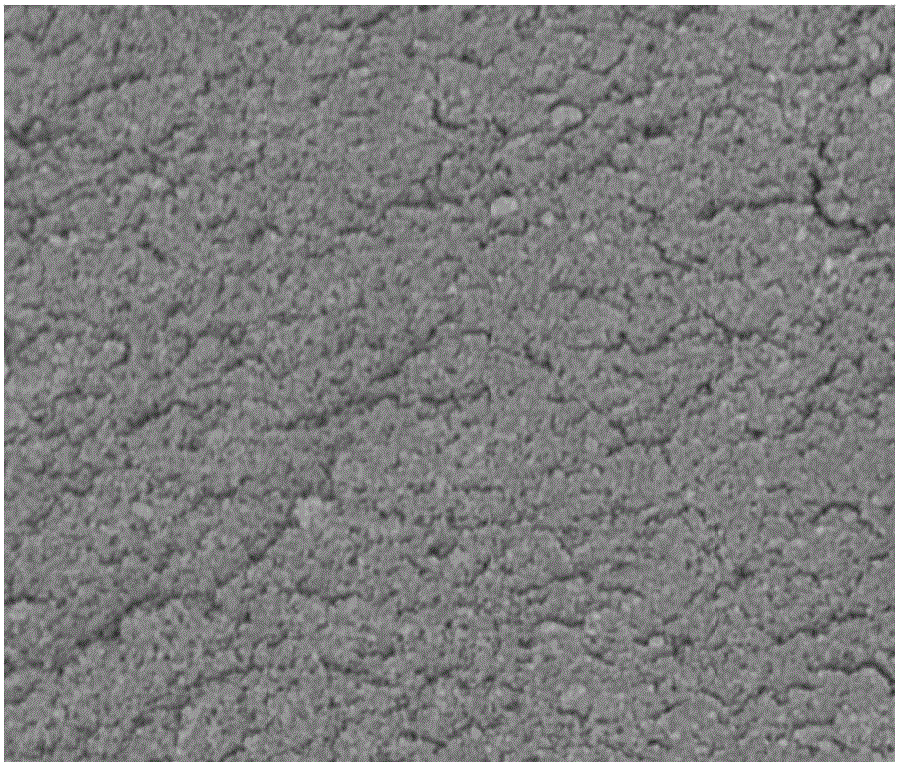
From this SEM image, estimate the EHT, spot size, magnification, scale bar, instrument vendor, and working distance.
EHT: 3 kV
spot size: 3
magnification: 80 K X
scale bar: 1000 nm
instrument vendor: FEI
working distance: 5 mm